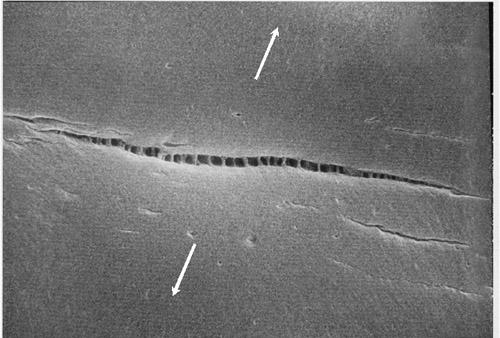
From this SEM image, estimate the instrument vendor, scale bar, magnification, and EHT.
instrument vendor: JEOL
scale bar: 10000 nm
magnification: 2 K X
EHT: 20 kV